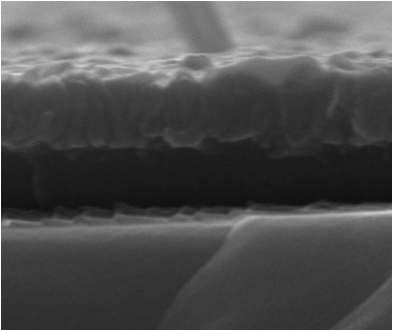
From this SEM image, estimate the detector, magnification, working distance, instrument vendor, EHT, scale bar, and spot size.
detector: ETD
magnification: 200 K X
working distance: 12.5 mm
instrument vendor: FEI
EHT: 20 kV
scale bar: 500 nm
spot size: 3